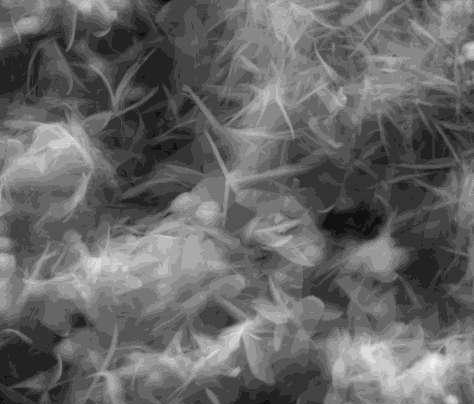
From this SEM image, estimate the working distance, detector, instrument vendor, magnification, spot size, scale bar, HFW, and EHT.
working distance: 4.8 mm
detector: LVD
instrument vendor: FEI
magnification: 10 K X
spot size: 4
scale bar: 10000 nm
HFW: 29.8 µm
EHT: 18 kV